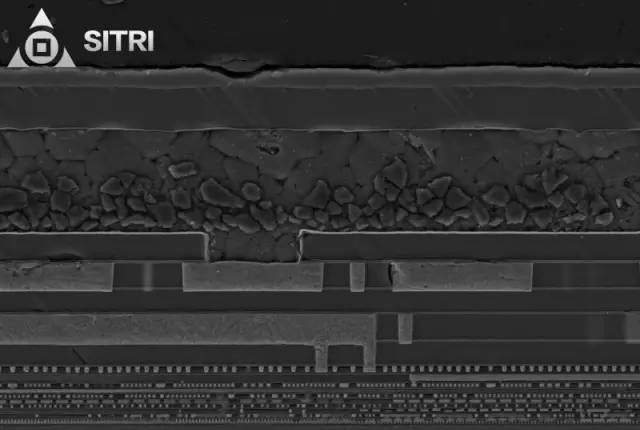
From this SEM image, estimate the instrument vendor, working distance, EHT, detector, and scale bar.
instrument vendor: Zeiss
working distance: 4.3 mm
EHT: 5 kV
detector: SE2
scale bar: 1000 nm